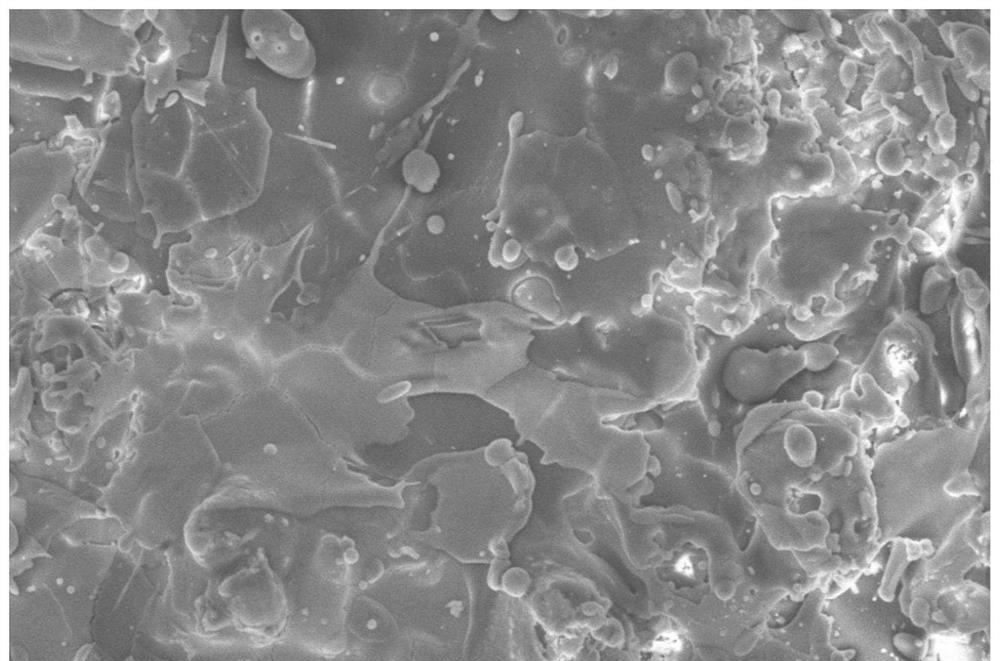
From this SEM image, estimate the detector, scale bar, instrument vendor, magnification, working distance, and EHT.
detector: SE(M)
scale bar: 50000 nm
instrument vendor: Hitachi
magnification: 1 K X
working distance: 14.8 mm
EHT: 15 kV